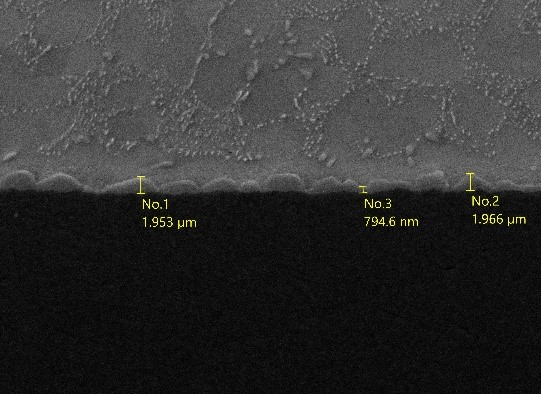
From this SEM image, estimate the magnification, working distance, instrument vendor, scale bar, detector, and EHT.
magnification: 2 K X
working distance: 10.2 mm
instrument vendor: JEOL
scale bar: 10000 nm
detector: SED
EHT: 20 kV